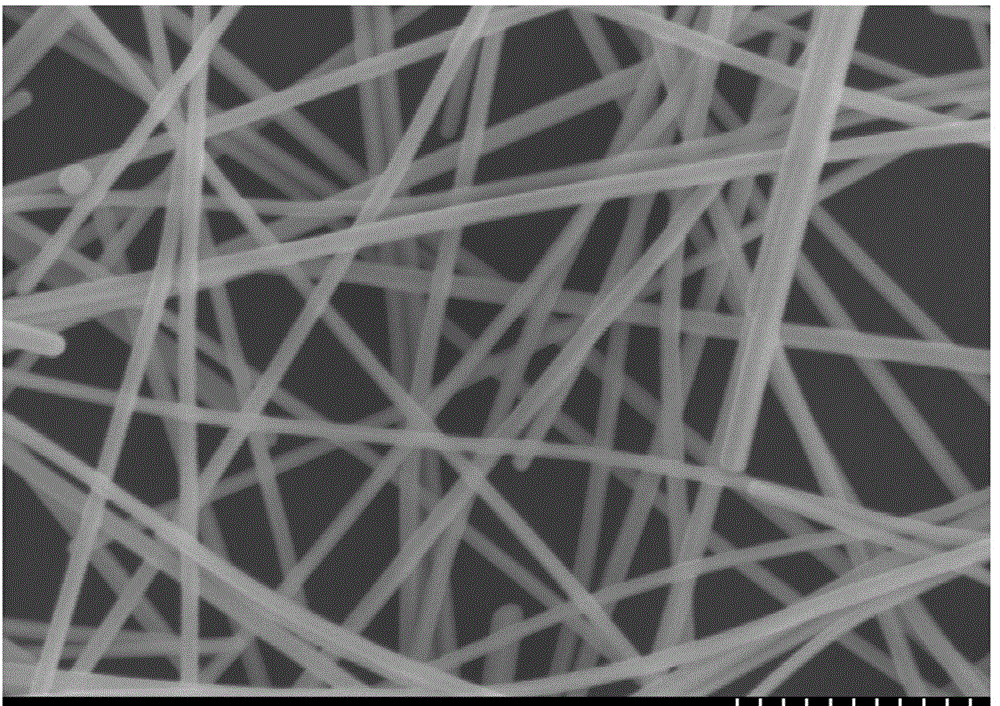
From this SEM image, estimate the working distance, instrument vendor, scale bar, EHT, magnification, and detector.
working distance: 8.9 mm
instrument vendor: Hitachi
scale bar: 500 nm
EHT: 5 kV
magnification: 60 K X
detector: SE(M)